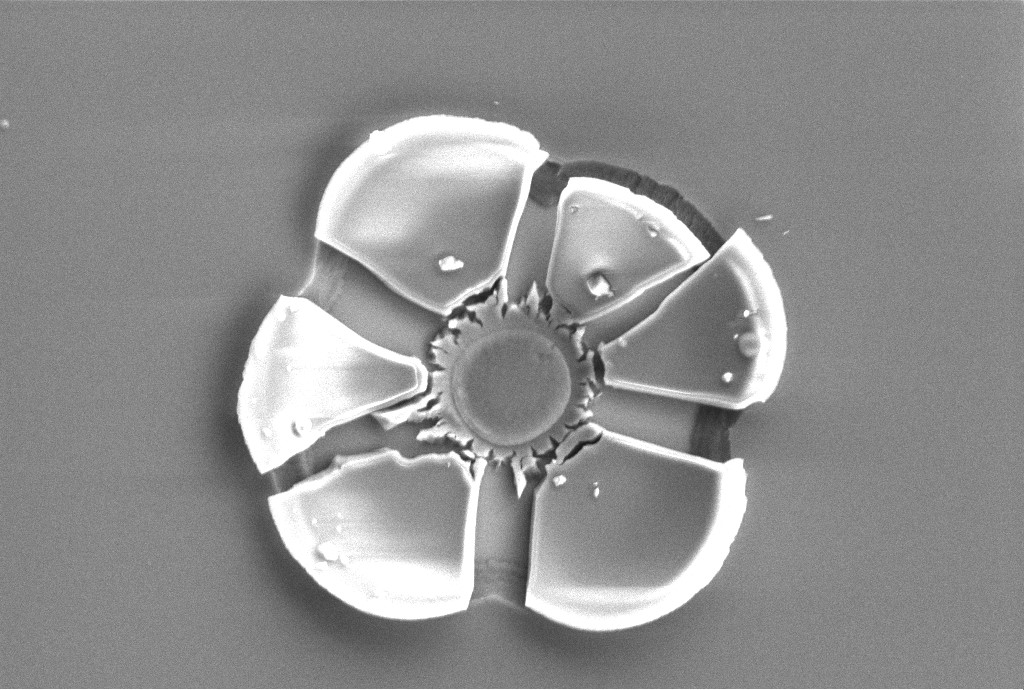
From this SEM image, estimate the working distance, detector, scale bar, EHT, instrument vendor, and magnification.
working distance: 10.6 mm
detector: SE2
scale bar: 1000 nm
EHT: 3 kV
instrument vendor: Zeiss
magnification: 6 K X